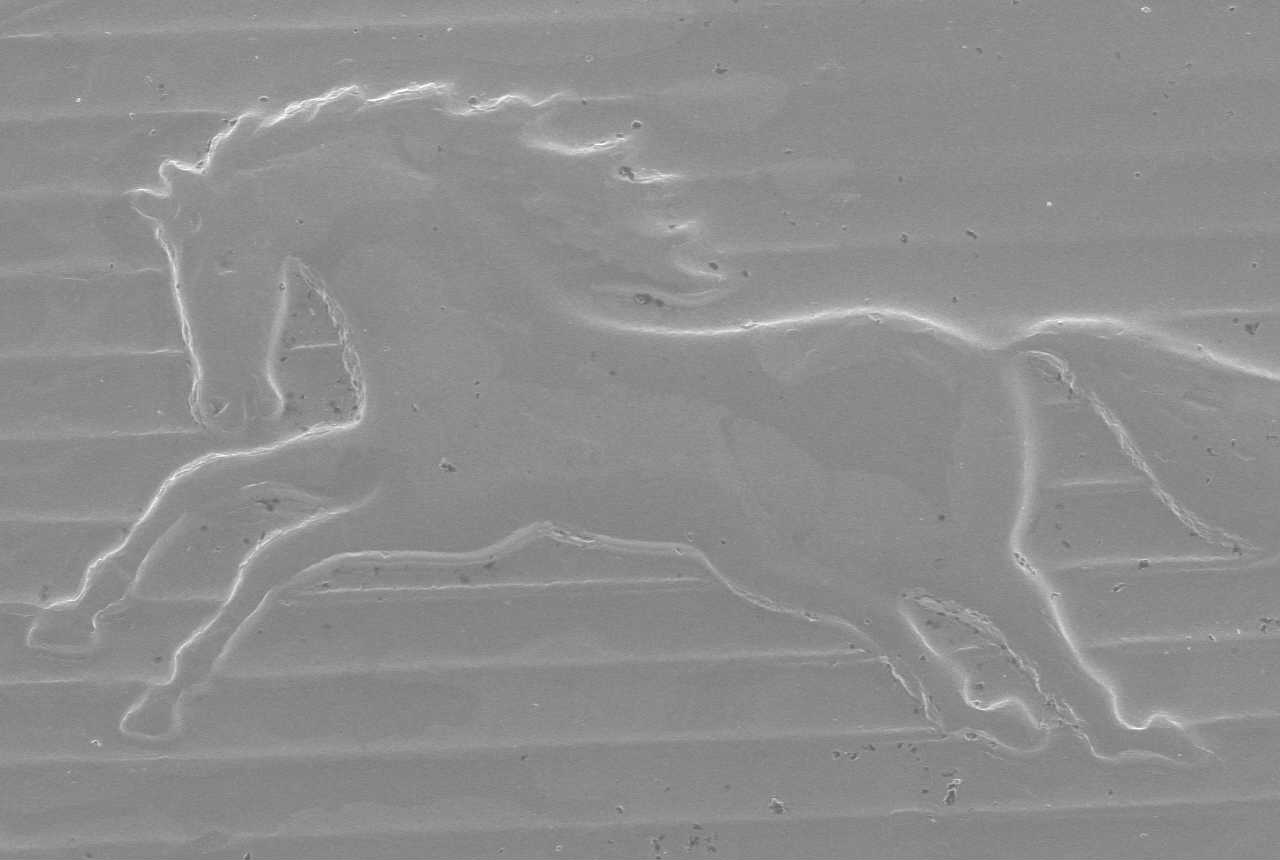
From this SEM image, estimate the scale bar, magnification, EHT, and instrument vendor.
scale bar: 1000000 nm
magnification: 0.022 K X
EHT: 20 kV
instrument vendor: JEOL